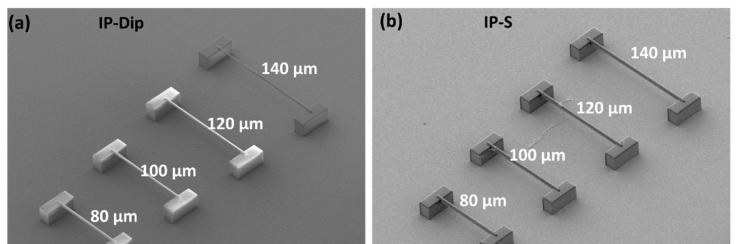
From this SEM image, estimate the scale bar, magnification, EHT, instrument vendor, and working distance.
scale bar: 100000 nm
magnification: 0.3 K X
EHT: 5 kV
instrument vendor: Hitachi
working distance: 29.5 mm